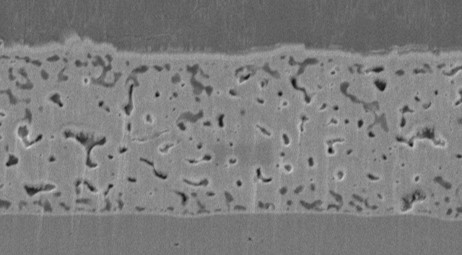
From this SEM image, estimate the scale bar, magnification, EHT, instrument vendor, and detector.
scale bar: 5000 nm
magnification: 5 K X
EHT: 10 kV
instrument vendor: JEOL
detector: SEI